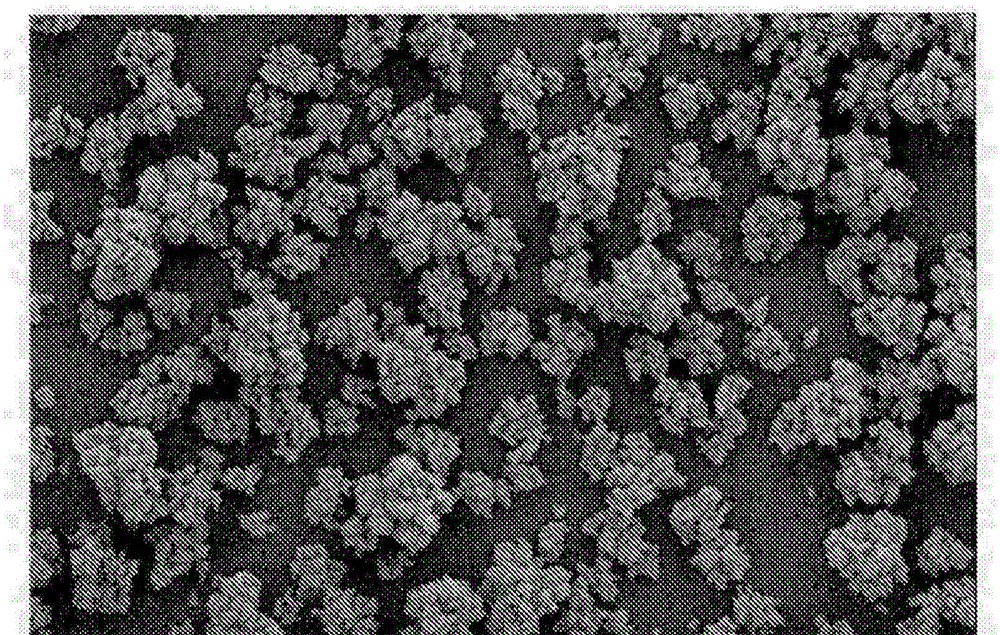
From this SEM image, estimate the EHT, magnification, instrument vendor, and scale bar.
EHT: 5 kV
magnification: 1 K X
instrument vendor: JEOL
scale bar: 10000 nm